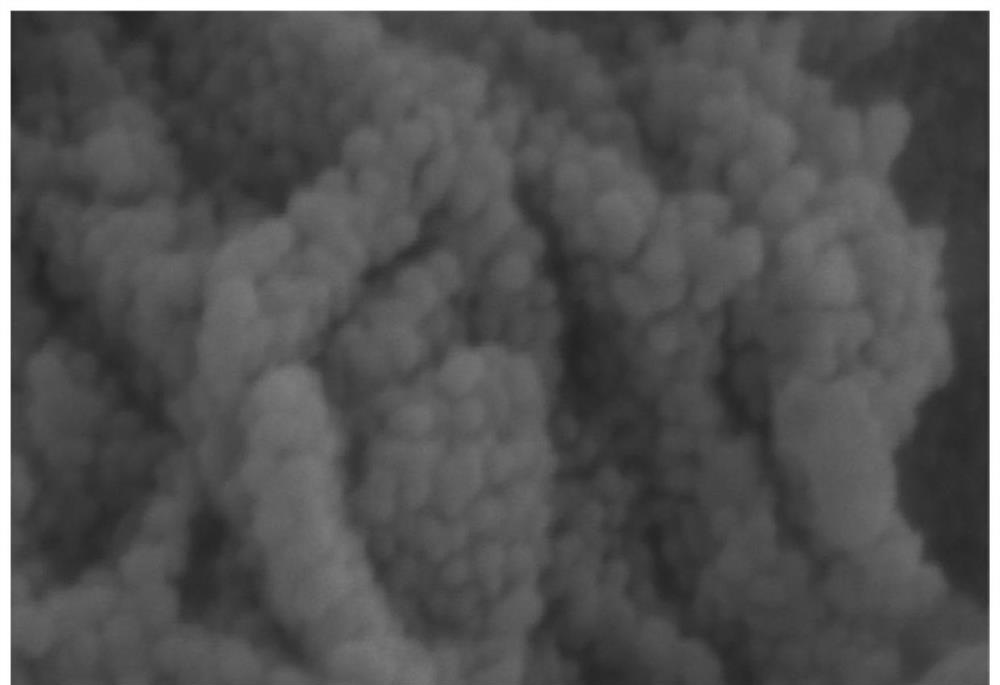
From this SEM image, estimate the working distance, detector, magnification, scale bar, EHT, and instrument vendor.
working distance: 10.6 mm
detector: SE(UL)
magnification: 200 K X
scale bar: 200 nm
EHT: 3 kV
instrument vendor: Hitachi